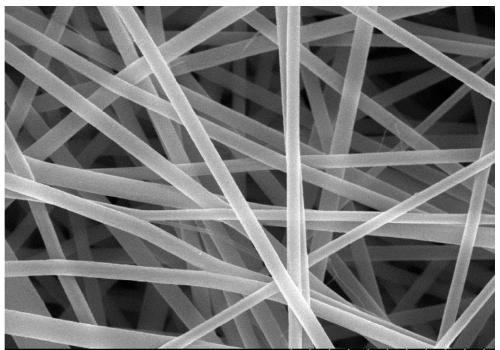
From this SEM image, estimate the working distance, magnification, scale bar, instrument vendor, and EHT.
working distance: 10.9 mm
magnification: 5 K X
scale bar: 10000 nm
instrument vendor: Hitachi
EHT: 20 kV